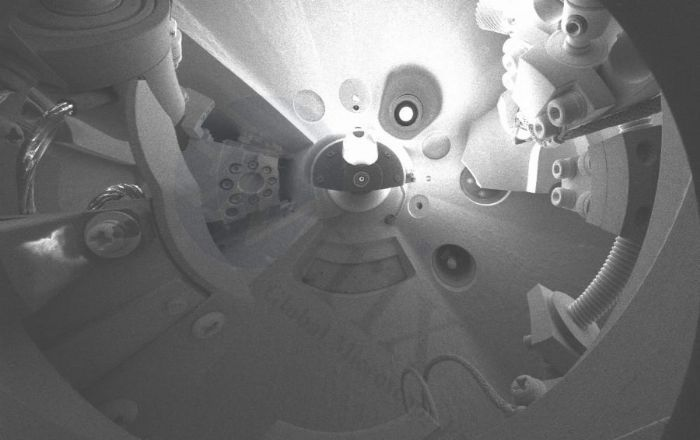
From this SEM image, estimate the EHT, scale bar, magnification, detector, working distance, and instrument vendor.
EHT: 5 kV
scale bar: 1000000 nm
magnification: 0.03 K X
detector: SE(M)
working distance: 12 mm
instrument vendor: Hitachi